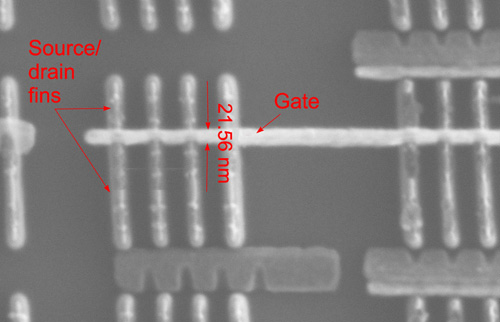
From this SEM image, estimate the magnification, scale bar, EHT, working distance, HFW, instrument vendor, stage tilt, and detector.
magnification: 250 K X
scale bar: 200 nm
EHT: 30 kV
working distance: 3.6 mm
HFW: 0.829 µm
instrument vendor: FEI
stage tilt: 0°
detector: ETD